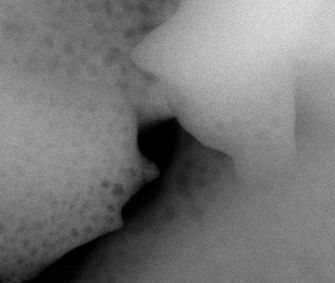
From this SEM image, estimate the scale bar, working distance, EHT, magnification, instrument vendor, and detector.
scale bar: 1000 nm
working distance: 10.1 mm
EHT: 30 kV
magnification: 50 K X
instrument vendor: FEI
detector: BSED